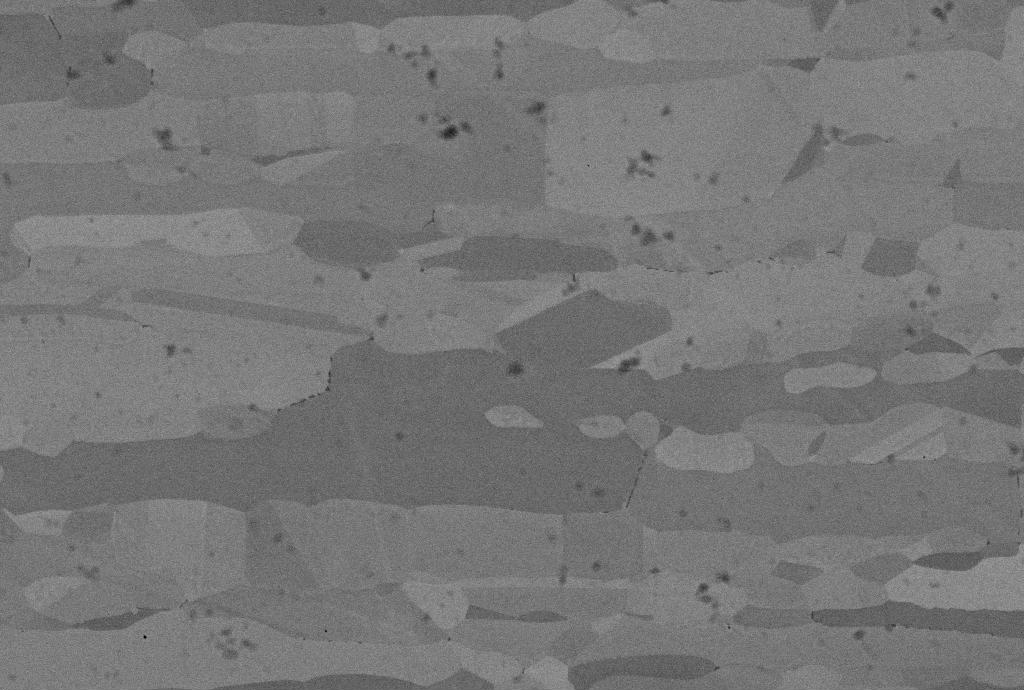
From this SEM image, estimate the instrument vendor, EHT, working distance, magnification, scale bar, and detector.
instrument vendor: Zeiss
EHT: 12.95 kV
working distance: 8 mm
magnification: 4.36 K X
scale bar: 10000 nm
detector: QBSD